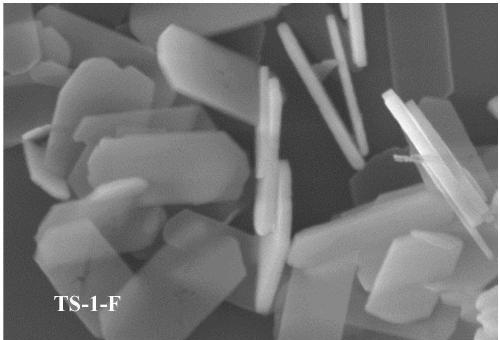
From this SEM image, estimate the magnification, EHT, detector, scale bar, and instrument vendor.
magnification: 120 K X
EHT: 5 kV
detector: SE(U)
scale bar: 1000 nm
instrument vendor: Hitachi